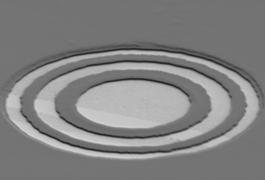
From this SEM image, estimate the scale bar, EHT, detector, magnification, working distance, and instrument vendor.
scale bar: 5000 nm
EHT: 1 kV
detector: SE(M)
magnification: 6.01 K X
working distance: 12 mm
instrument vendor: Hitachi